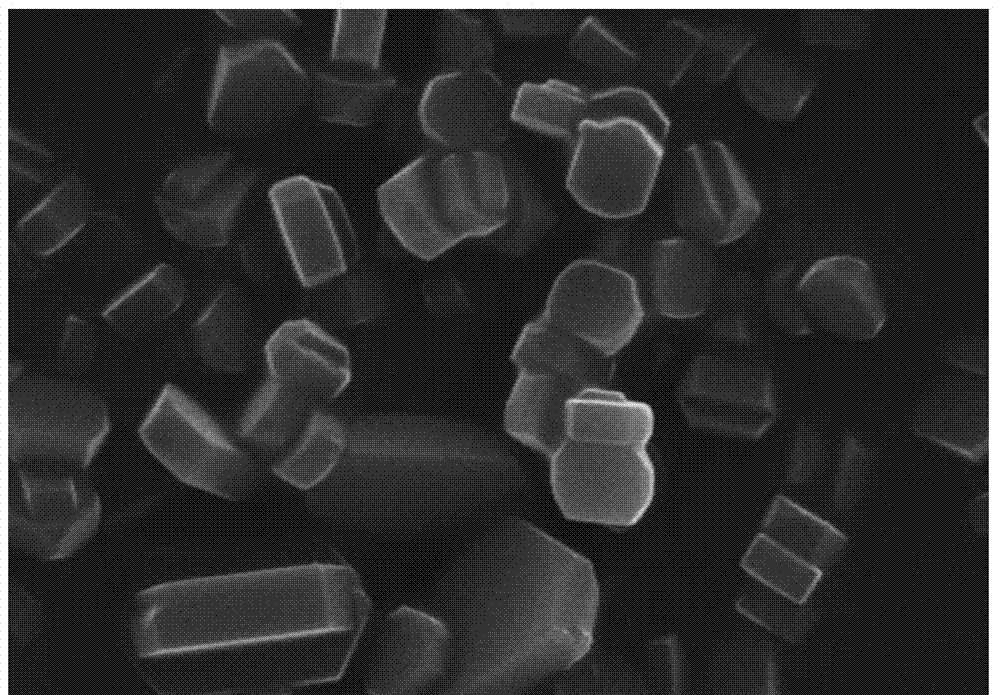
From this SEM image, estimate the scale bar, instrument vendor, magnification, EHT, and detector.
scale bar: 3000 nm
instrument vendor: Hitachi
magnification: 15 K X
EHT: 15 kV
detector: SE